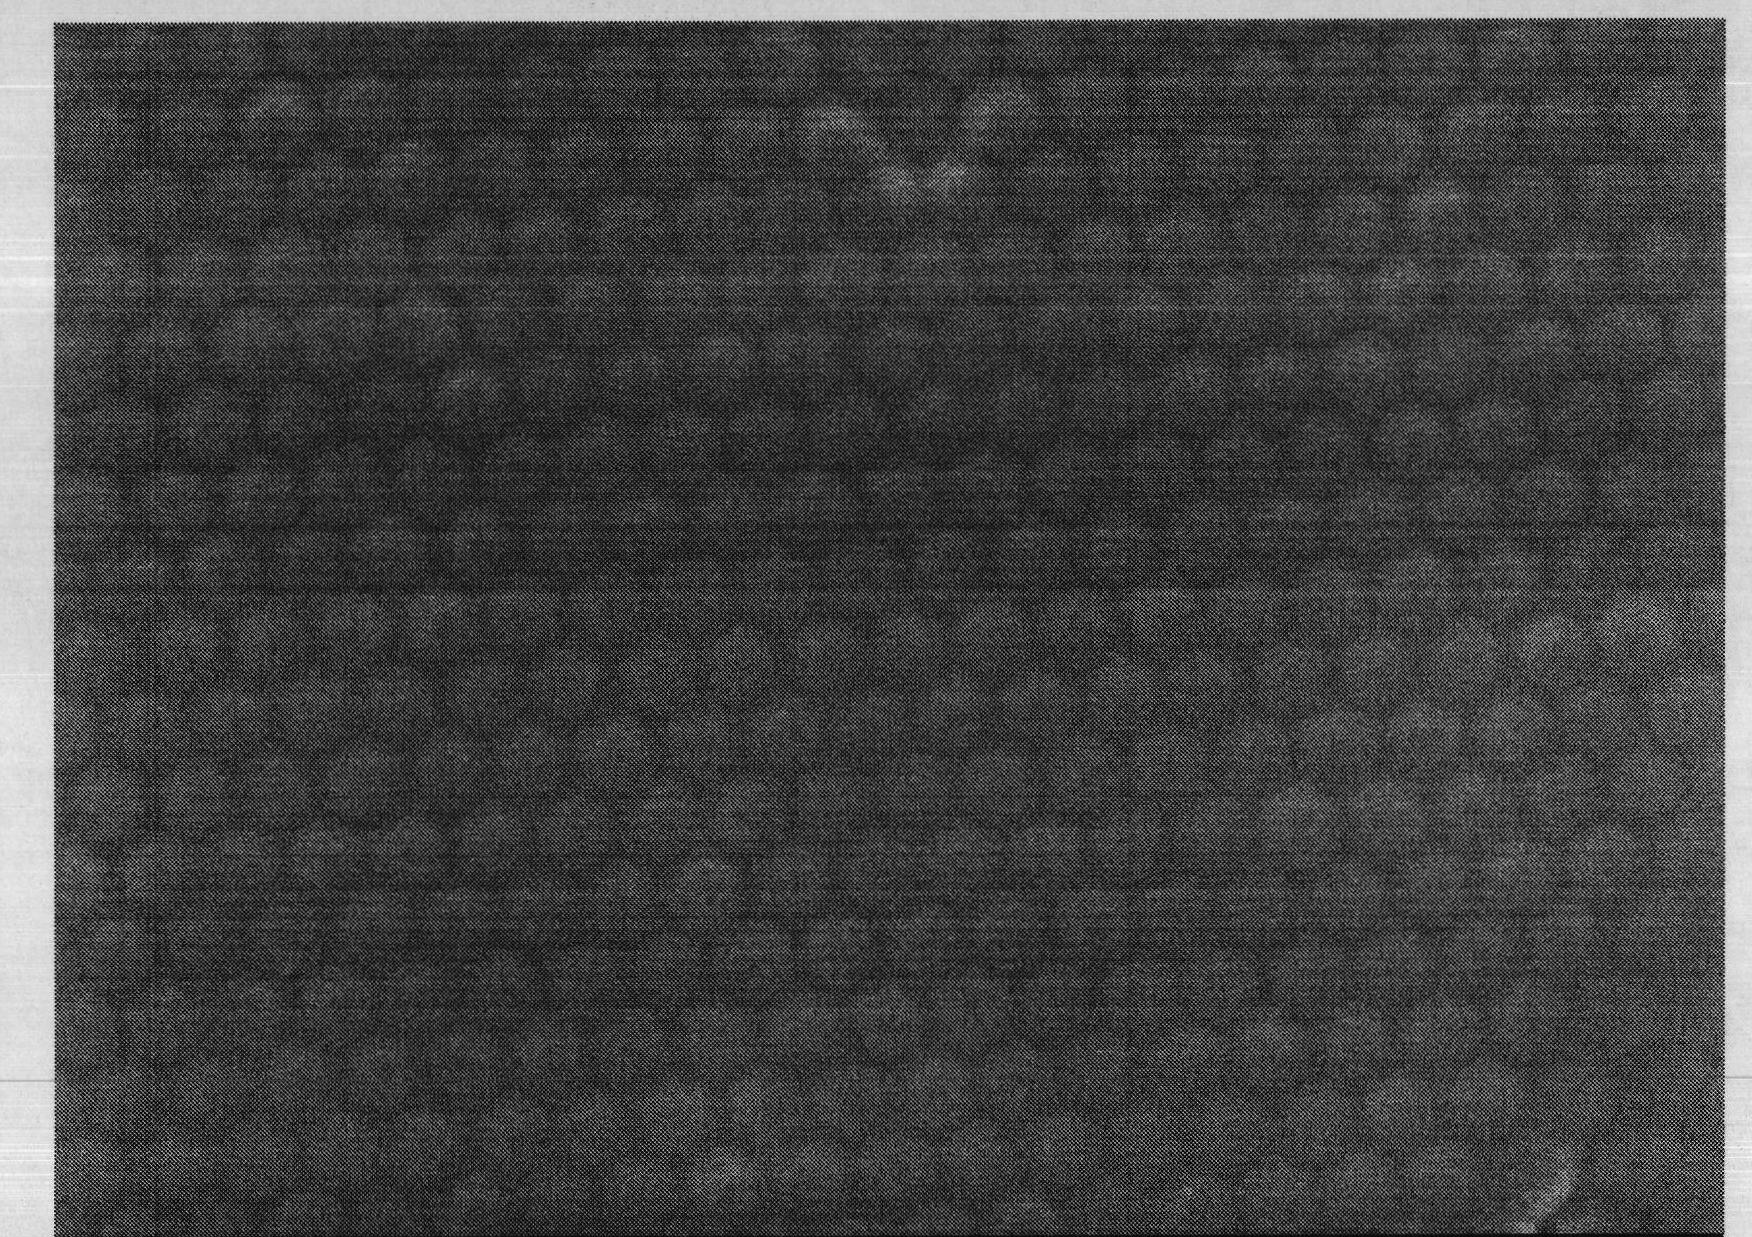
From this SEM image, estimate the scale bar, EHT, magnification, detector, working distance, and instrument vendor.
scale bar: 1000 nm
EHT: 3 kV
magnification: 20 K X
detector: SEI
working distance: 4.8 mm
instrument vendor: JEOL